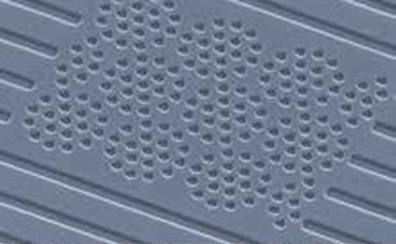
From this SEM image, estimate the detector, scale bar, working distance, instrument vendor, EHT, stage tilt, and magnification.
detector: SE2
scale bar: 1000 nm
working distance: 8 mm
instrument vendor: Zeiss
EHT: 20 kV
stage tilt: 44.6°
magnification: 14.19 K X